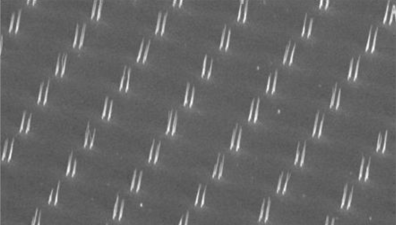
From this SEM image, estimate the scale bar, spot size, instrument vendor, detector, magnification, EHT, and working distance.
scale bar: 10000 nm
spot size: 3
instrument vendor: FEI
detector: SE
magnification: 1.976 K X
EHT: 5 kV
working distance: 26.4 mm